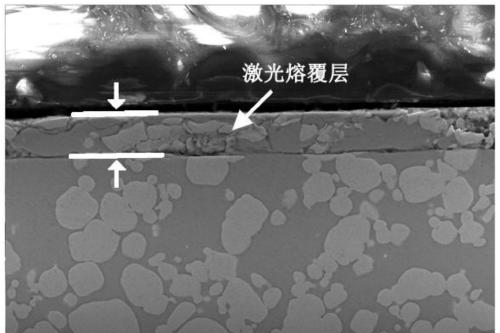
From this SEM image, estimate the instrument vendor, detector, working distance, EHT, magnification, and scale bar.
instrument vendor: Zeiss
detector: InLens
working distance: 10.3 mm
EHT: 20 kV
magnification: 1 K X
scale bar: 10000 nm